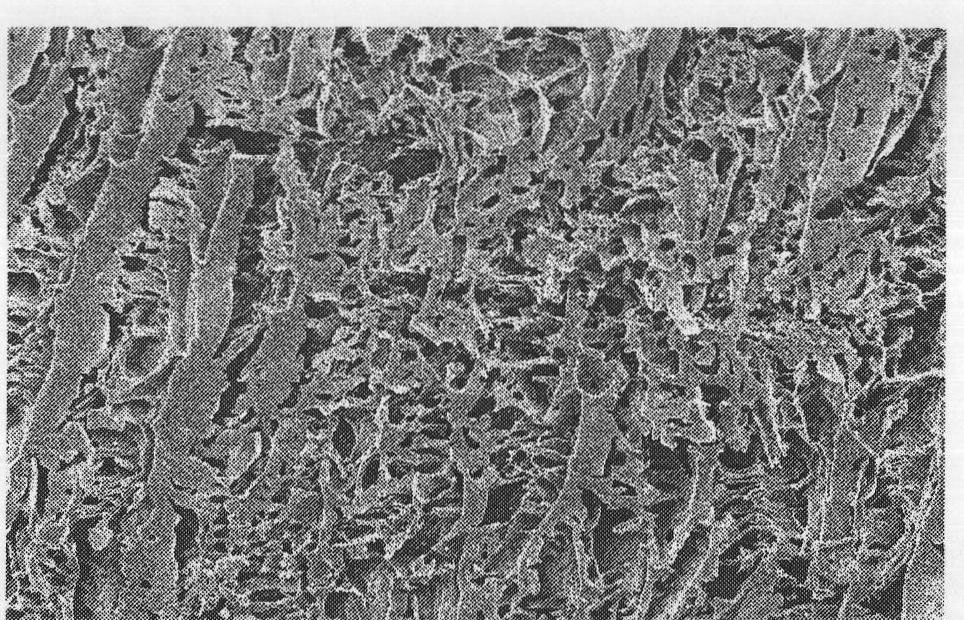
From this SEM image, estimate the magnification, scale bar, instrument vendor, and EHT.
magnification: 0.05 K X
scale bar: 500000 nm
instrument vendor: JEOL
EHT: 15 kV